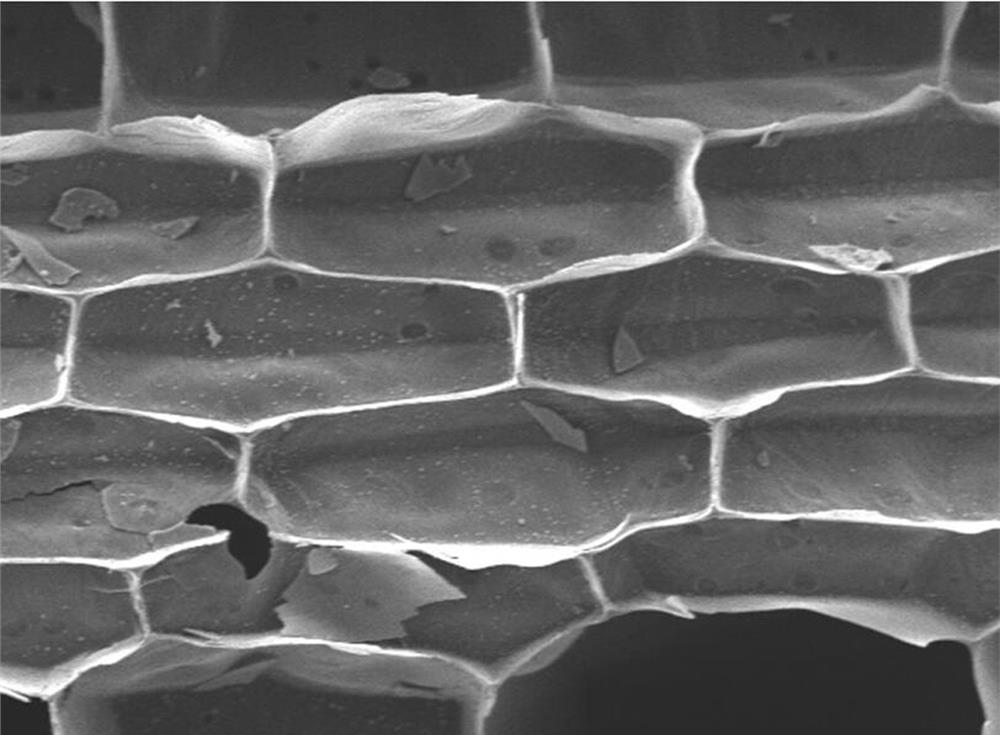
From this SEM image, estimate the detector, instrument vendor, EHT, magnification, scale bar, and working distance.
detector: LED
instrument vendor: JEOL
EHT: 5 kV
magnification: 1 K X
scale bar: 10000 nm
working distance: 9.1 mm